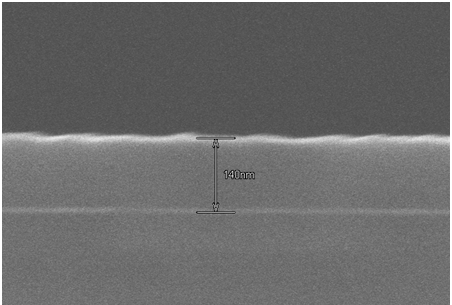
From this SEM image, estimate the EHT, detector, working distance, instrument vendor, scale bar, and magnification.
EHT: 15 kV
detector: SE(U)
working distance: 13.8 mm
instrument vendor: Hitachi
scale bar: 300 nm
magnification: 150 K X